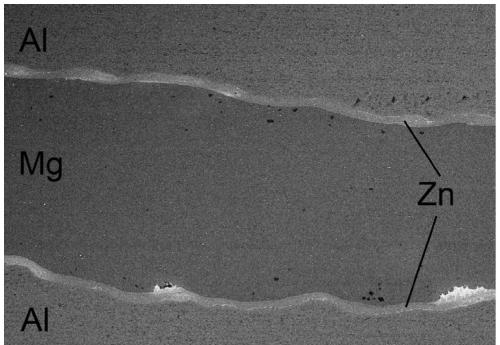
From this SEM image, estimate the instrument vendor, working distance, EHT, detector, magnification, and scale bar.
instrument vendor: Hitachi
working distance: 18.5 mm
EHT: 16 kV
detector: BSECOMP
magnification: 0.1 K X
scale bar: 500000 nm